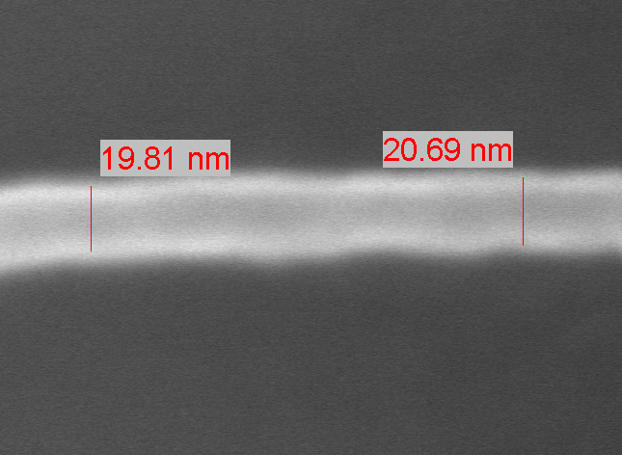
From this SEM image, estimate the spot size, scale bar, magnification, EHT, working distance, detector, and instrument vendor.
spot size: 2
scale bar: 20 nm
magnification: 650 K X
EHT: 5 kV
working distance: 4.9 mm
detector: TLD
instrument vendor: FEI